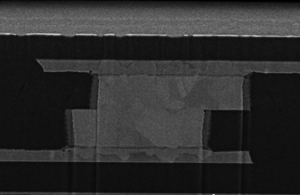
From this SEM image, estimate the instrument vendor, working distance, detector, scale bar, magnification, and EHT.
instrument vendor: Hitachi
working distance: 4 mm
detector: SE(U,LA50)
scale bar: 2000 nm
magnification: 20 K X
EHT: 3 kV